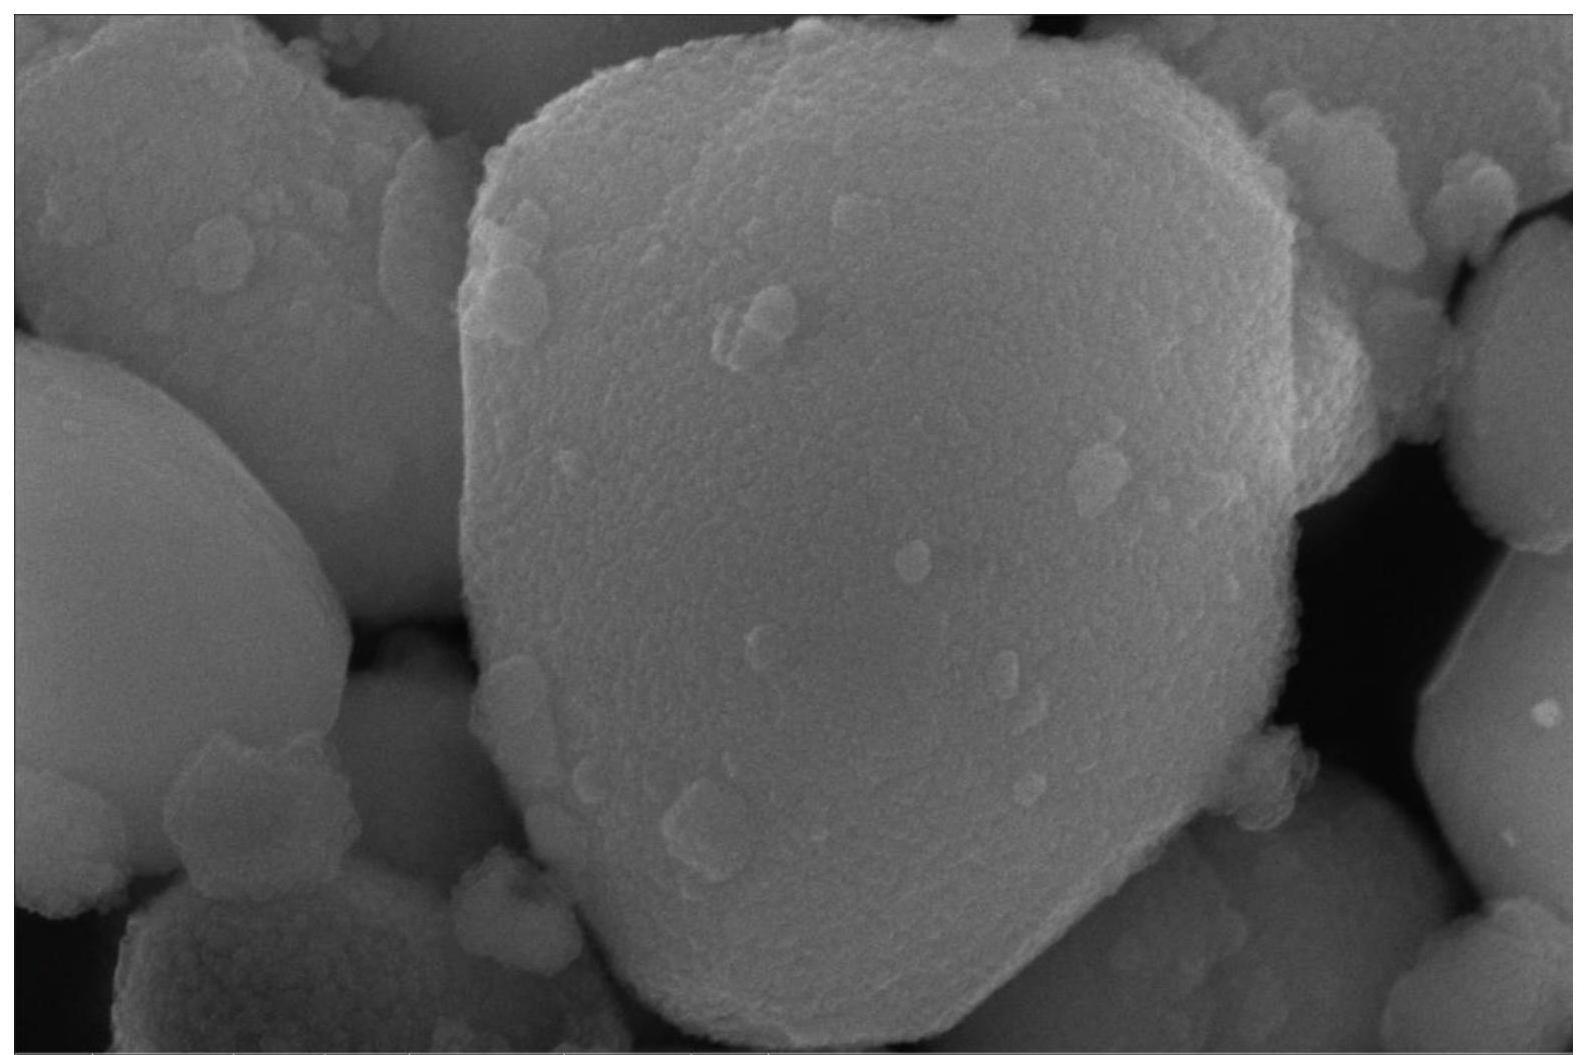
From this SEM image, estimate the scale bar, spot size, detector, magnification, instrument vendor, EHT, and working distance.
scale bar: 500 nm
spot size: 3.5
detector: TLD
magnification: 200 K X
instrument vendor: FEI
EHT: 20 kV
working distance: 6.5 mm